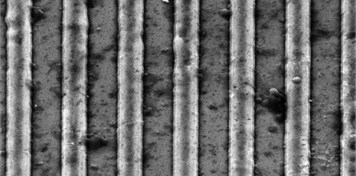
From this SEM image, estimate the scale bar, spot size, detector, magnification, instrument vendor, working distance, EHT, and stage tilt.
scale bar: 10000 nm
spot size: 3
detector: ETD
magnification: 10 K X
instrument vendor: FEI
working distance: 10.1 mm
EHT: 20 kV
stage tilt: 45°